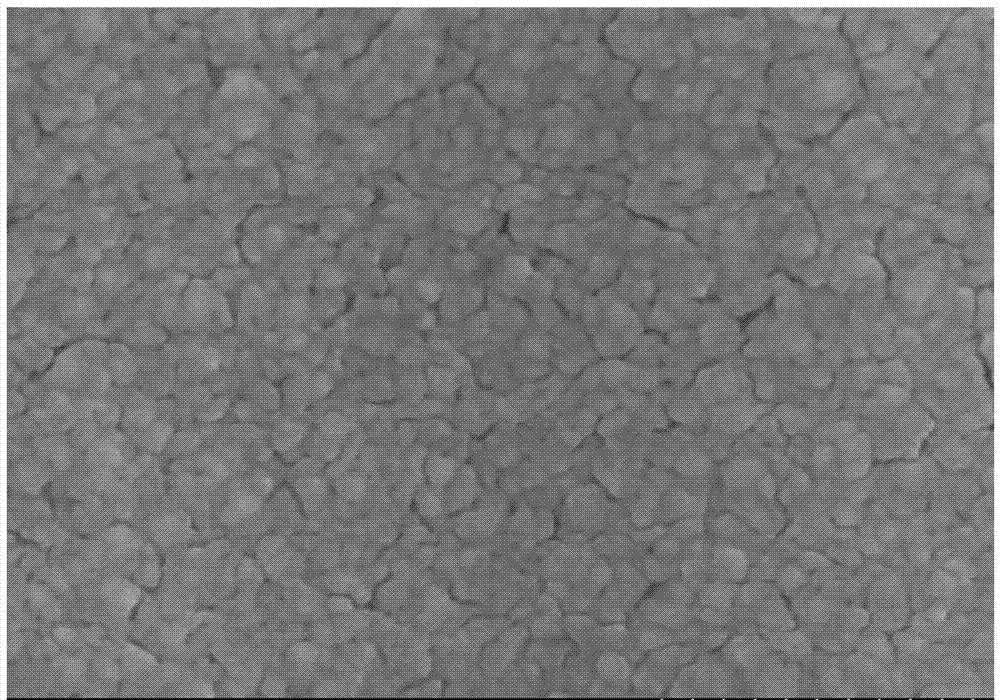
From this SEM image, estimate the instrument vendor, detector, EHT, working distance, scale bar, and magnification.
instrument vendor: Hitachi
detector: SE(M)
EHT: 20 kV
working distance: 11.8 mm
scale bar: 500 nm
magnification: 80 K X